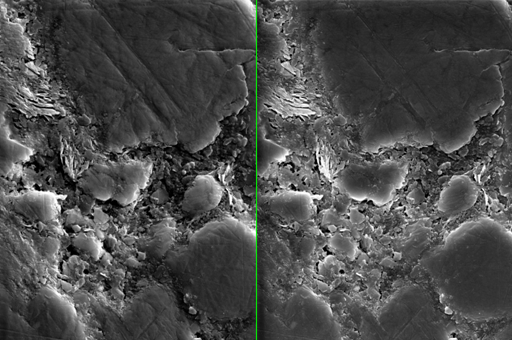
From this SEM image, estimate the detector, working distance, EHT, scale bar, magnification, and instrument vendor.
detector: InLens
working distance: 9.3 mm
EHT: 5 kV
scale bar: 1000 nm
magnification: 13 K X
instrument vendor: Zeiss